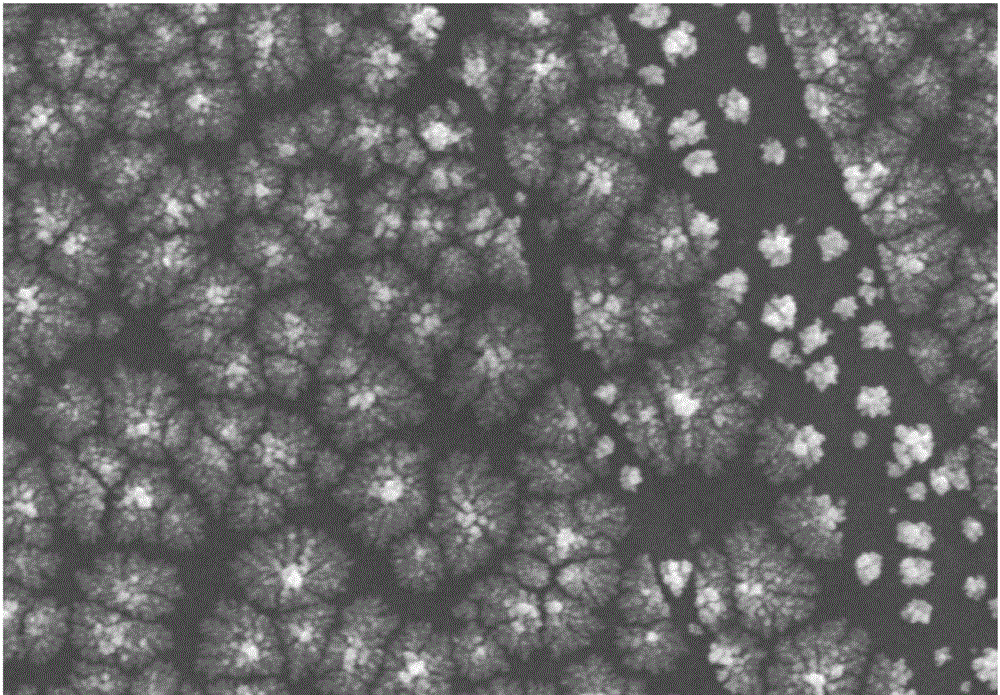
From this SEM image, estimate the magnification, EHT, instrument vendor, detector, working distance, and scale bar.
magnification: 15 K X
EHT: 15 kV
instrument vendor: Hitachi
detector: SE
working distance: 5 mm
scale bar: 3000 nm